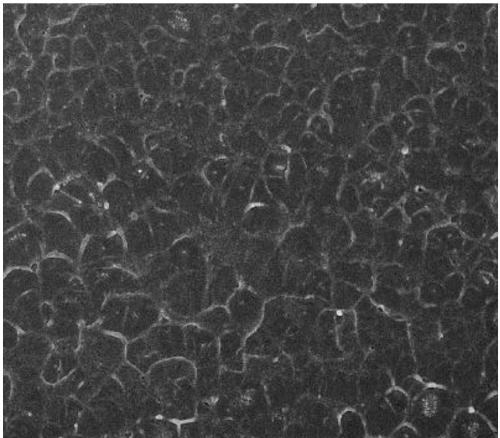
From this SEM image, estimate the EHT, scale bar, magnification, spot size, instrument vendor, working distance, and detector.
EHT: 10 kV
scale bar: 5000 nm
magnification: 10 K X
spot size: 4.5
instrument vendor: FEI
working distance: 5.6 mm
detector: TLD